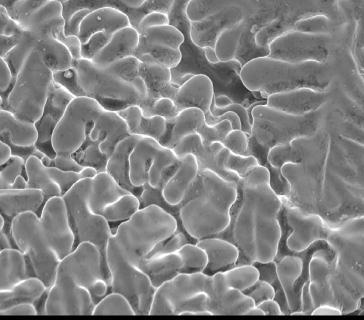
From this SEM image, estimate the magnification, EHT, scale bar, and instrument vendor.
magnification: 0.4 K X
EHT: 15 kV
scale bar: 50000 nm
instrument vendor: JEOL